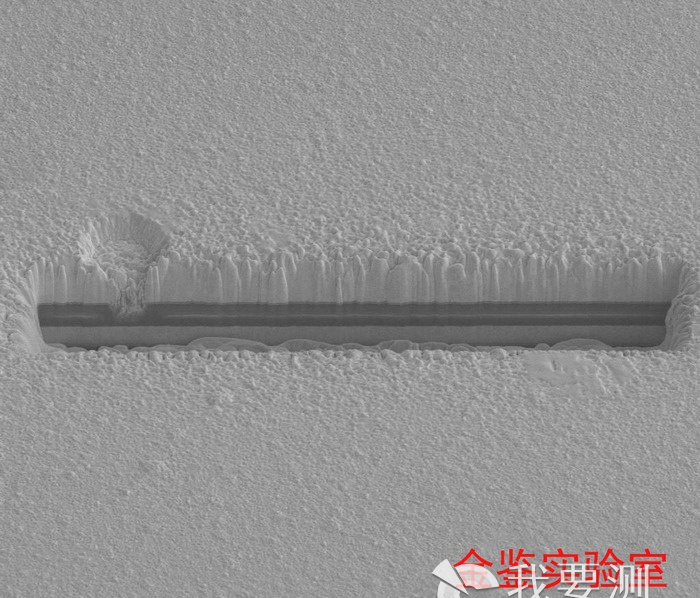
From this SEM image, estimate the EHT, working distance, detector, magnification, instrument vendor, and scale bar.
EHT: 5 kV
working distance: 4.9 mm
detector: ETD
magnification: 10 K X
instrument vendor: FEI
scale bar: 10000 nm